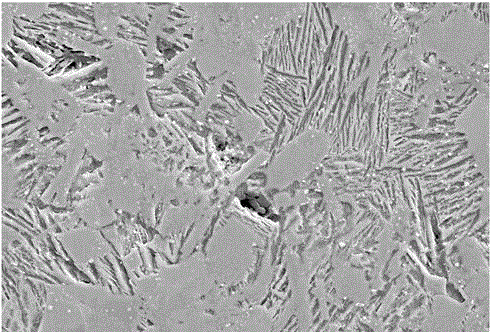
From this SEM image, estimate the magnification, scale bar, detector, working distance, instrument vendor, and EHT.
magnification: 1 K X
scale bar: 10000 nm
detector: SE2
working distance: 9.7 mm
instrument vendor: Zeiss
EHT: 15 kV